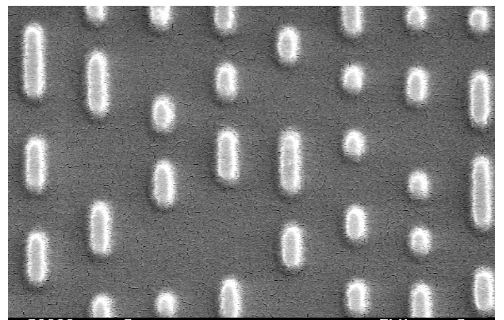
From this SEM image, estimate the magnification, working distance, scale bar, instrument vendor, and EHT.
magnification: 20 K X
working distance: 6 mm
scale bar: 2000 nm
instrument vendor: JEOL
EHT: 5 kV